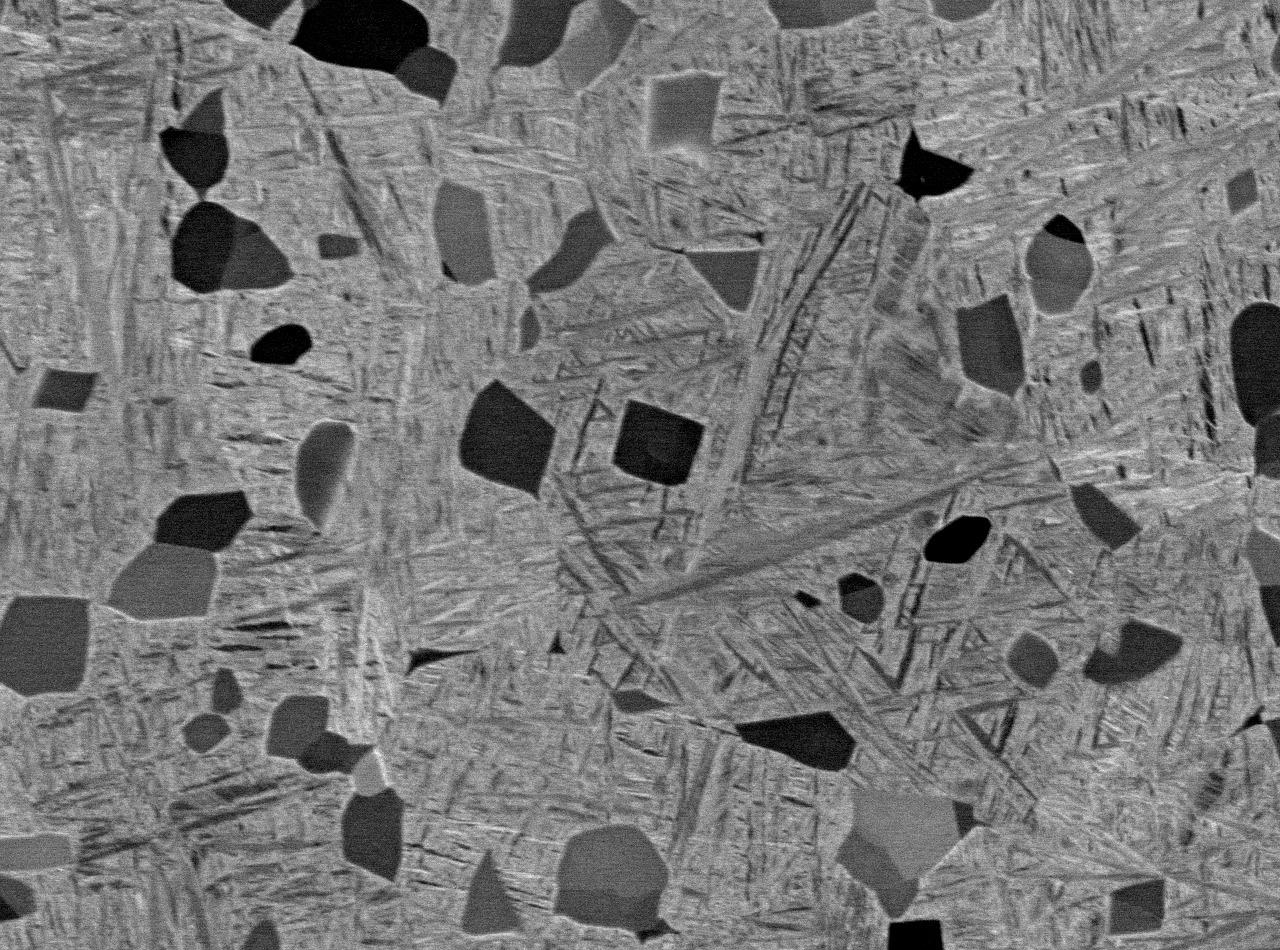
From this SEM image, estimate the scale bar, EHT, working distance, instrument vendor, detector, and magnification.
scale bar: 10000 nm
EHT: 15 kV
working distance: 10 mm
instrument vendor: JEOL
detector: COMPO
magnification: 1.5 K X